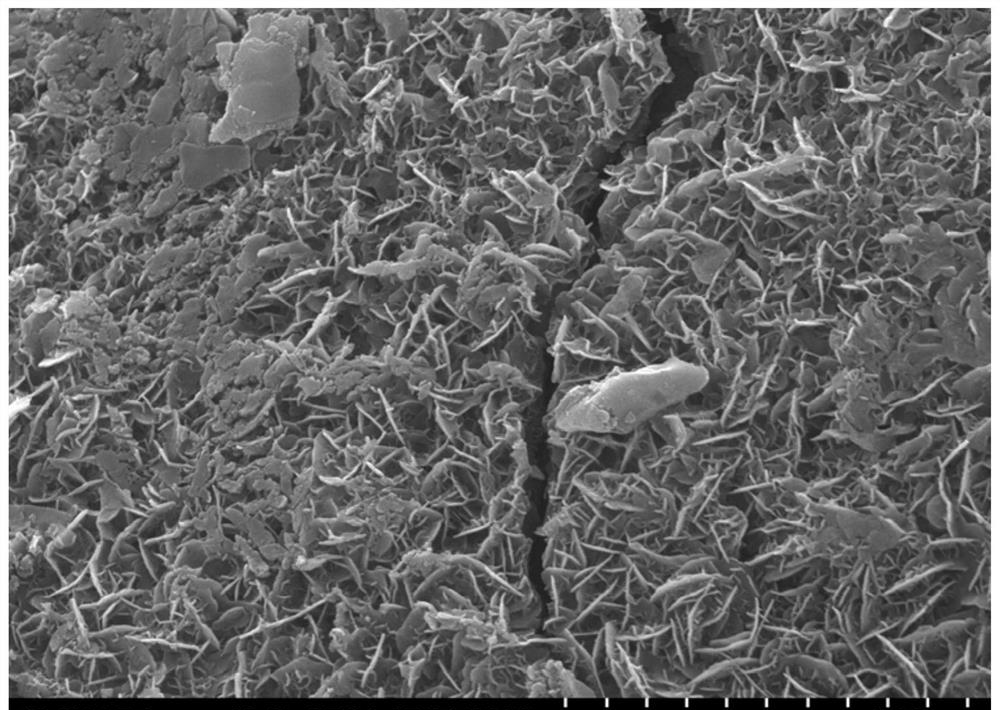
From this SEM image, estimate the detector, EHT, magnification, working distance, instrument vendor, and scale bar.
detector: SE(L)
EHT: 15 kV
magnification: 13 K X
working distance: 5.6 mm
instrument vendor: Hitachi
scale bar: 4000 nm